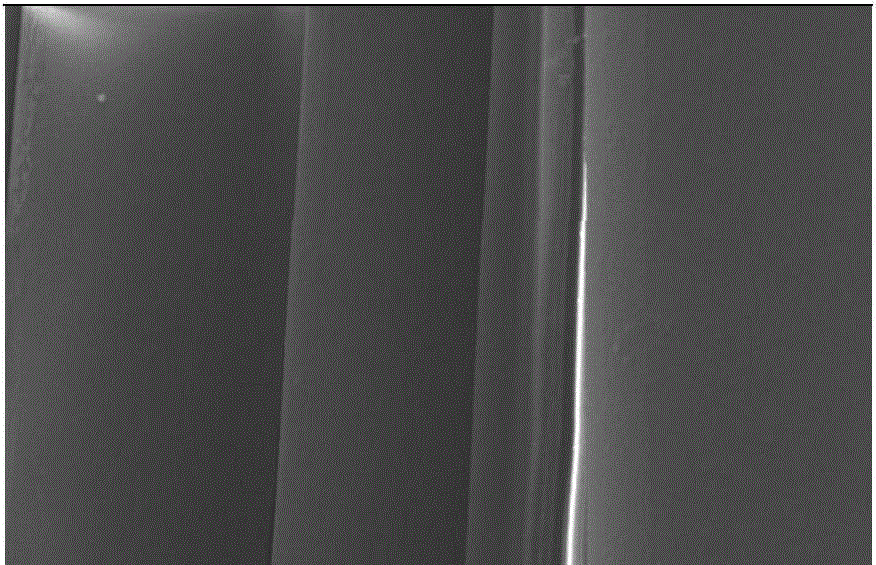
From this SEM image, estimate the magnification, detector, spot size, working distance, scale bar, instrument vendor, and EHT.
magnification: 2 K X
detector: SE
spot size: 3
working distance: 7.9 mm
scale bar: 10000 nm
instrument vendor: FEI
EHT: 10 kV